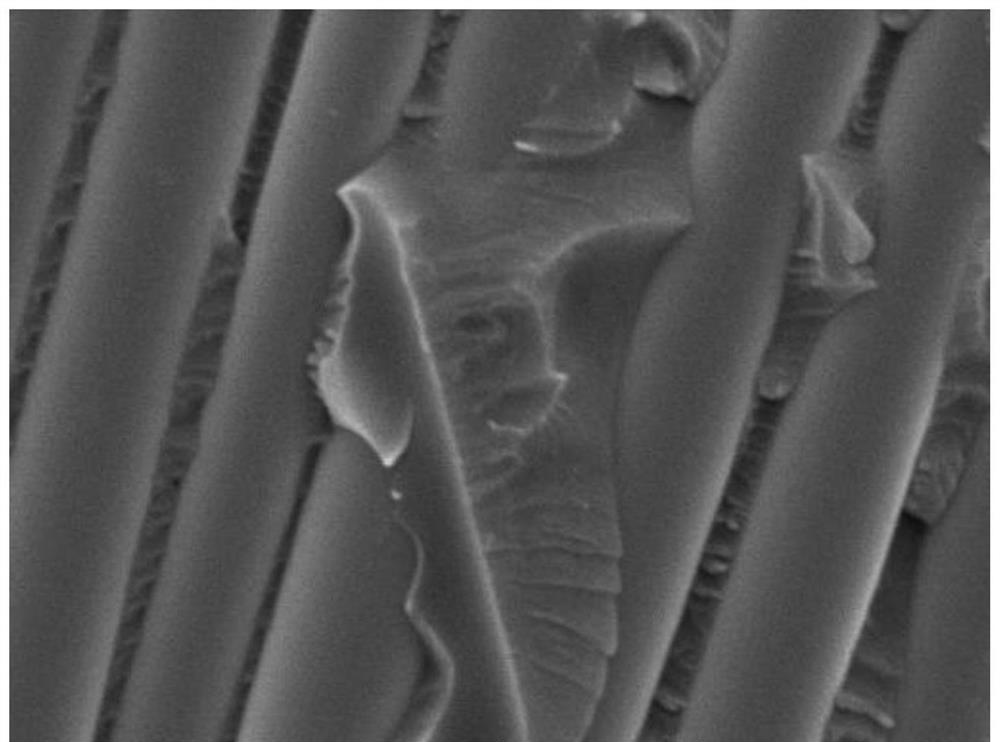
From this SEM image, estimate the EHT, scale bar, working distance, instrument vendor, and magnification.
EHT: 15 kV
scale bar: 10000 nm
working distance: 10.67 mm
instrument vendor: Tescan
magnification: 3 K X